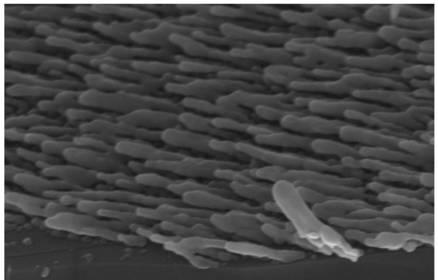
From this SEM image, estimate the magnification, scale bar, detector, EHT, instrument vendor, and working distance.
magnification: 50 K X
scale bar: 100 nm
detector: InlensDuo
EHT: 15 kV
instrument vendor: Zeiss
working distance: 7.5 mm